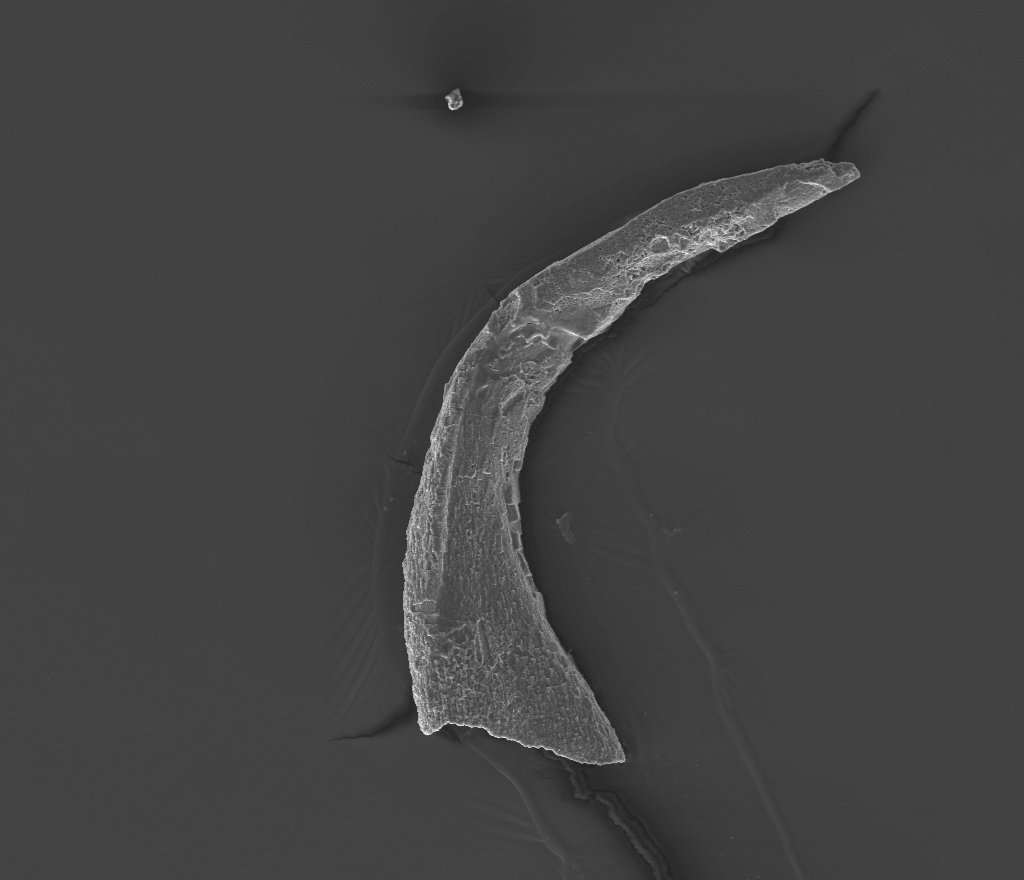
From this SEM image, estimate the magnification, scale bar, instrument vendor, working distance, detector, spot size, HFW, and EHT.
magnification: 0.3 K X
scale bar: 200000 nm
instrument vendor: FEI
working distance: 10 mm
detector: ETD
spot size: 5.5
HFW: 900 µm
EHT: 20 kV